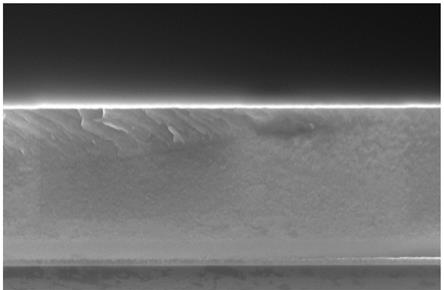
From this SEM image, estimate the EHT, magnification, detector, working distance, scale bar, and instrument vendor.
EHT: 10 kV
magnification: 15 K X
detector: TLD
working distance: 4 mm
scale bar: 2000 nm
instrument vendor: Thermo